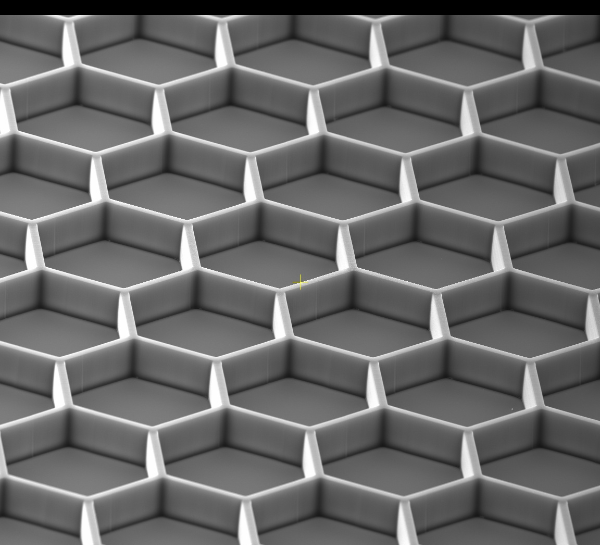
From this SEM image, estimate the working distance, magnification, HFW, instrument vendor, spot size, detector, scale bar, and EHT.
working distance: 13.8 mm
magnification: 0.5 K X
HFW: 829 µm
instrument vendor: FEI/Thermo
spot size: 12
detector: ETD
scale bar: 200000 nm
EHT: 30 kV